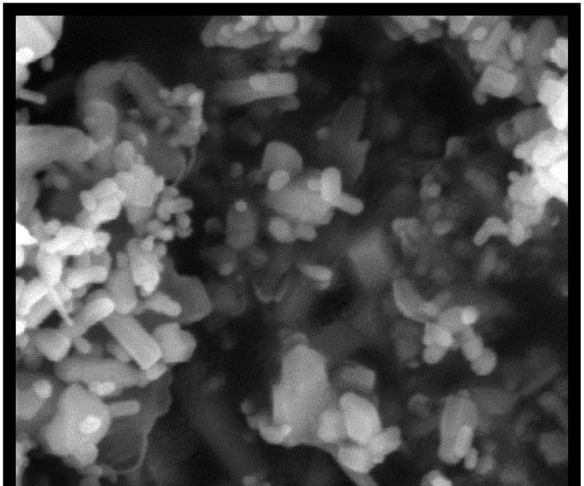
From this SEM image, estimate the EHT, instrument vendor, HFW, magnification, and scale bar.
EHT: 10 kV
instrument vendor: FEI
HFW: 1.86 µm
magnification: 80 K X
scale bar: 500 nm